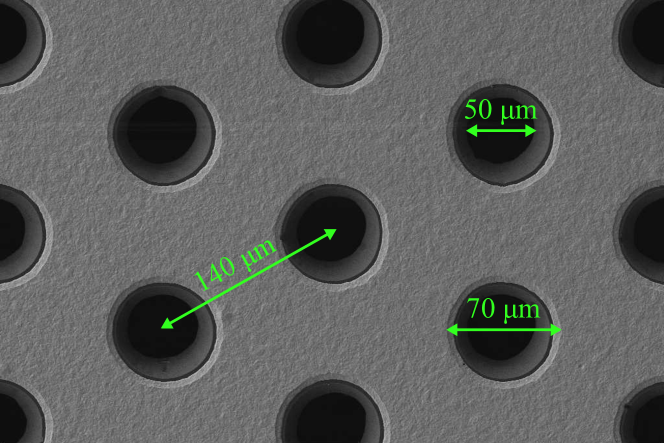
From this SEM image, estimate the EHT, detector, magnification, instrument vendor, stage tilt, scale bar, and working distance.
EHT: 5 kV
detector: SE2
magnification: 0.248 K X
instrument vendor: Zeiss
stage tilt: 0°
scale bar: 20000 nm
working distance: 5.1 mm